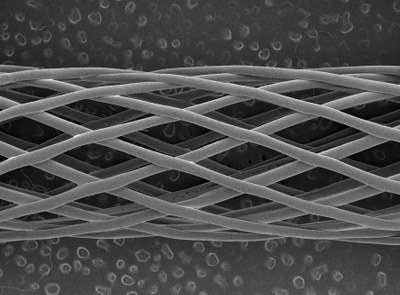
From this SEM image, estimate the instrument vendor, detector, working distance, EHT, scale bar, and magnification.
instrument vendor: JEOL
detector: SEI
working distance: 39 mm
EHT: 2 kV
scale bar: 1000000 nm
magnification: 0.015 K X